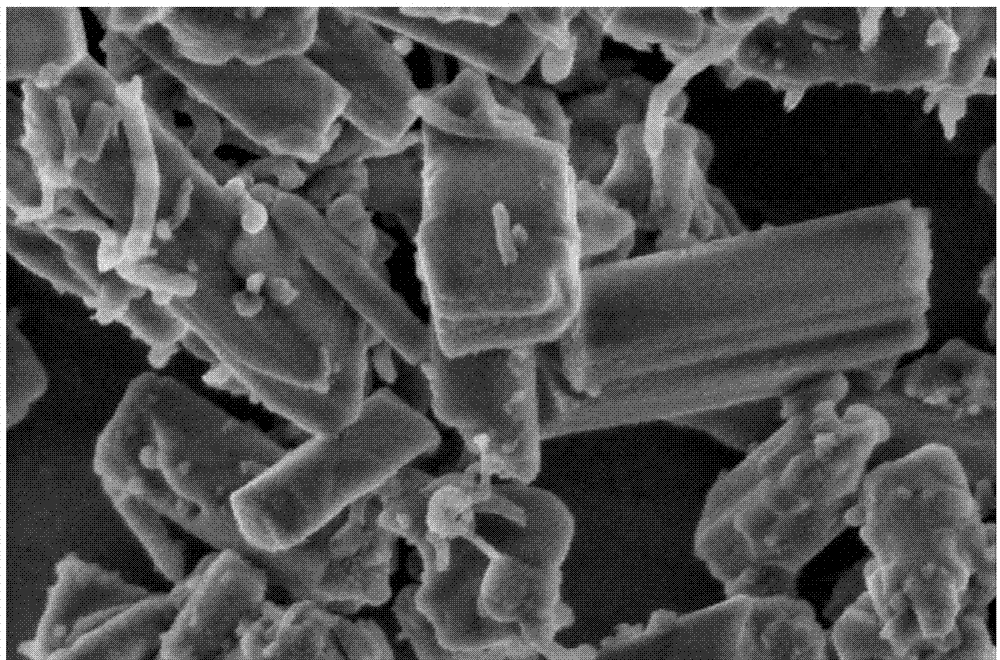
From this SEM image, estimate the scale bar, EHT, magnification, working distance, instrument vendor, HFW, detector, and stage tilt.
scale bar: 400 nm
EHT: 2 kV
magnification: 200 K X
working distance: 4.1 mm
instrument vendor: FEI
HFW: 2.07 µm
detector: TLD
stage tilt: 0°